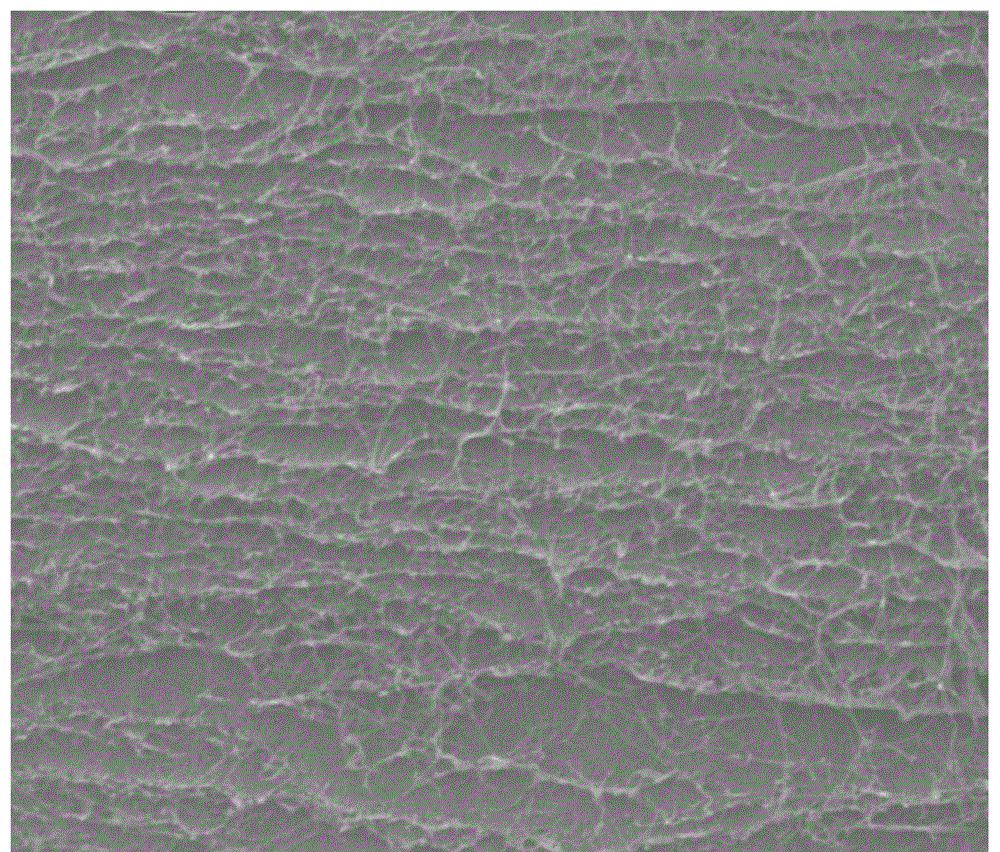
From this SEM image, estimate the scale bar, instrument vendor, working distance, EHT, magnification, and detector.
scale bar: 10000 nm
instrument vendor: FEI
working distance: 14.8 mm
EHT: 20 kV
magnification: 10 K X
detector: ETD